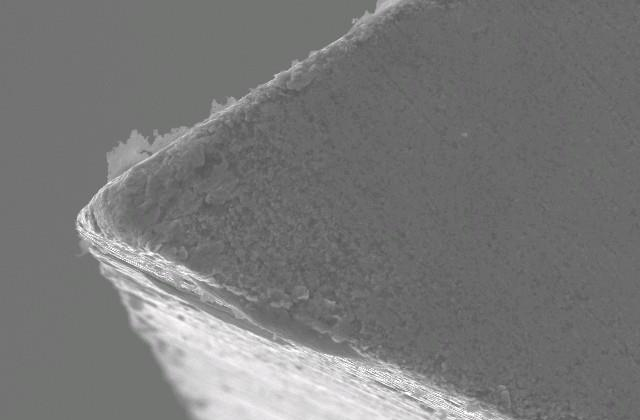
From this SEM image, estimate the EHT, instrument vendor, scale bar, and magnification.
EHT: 20 kV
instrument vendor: JEOL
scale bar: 10000 nm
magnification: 1 K X